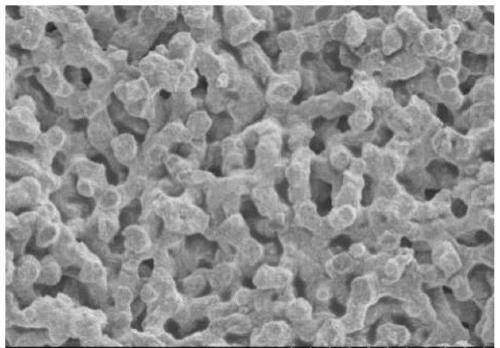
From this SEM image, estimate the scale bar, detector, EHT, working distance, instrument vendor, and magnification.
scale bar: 10000 nm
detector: SE(M)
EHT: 3 kV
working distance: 10 mm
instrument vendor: Hitachi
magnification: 5 K X